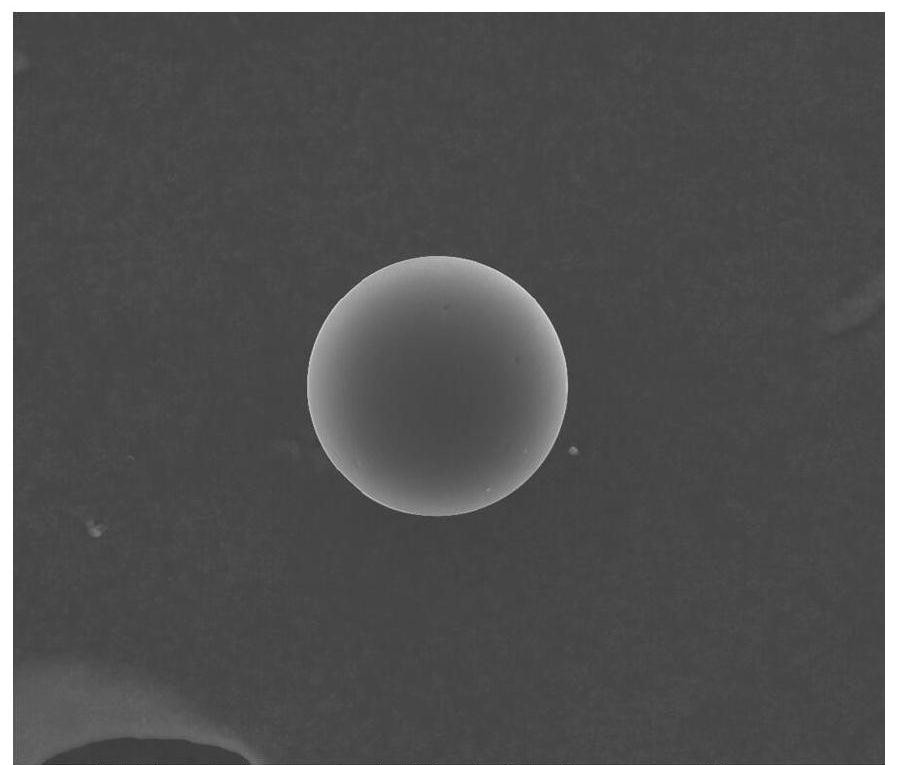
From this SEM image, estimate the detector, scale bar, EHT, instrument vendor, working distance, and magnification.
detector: ETD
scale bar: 100000 nm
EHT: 20 kV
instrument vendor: FEI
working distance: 11.4 mm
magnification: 1 K X